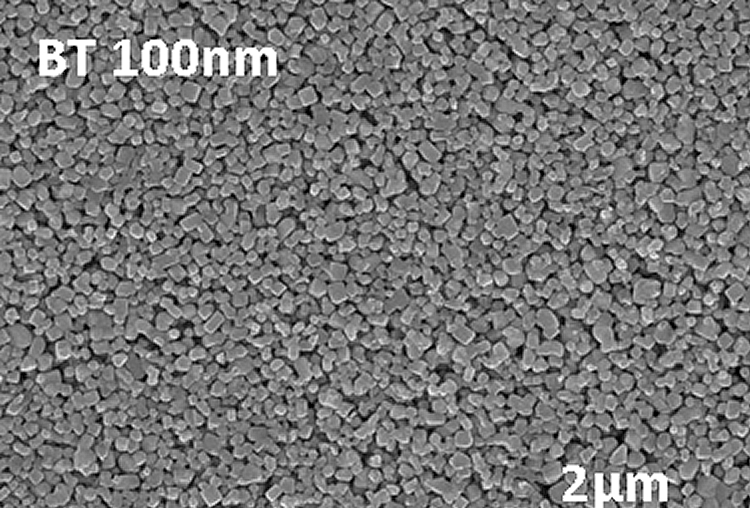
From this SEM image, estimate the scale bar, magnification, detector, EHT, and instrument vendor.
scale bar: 2000 nm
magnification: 20 K X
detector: SE(U)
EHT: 10 kV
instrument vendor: Hitachi